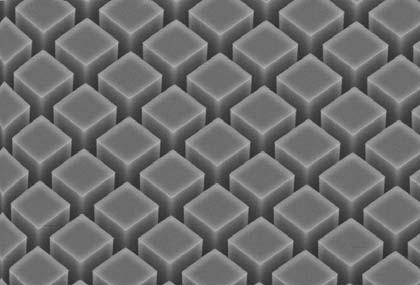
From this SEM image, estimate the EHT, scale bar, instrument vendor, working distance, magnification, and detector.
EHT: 25 kV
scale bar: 40000 nm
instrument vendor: JEOL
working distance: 48 mm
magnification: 0.45 K X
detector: SEI